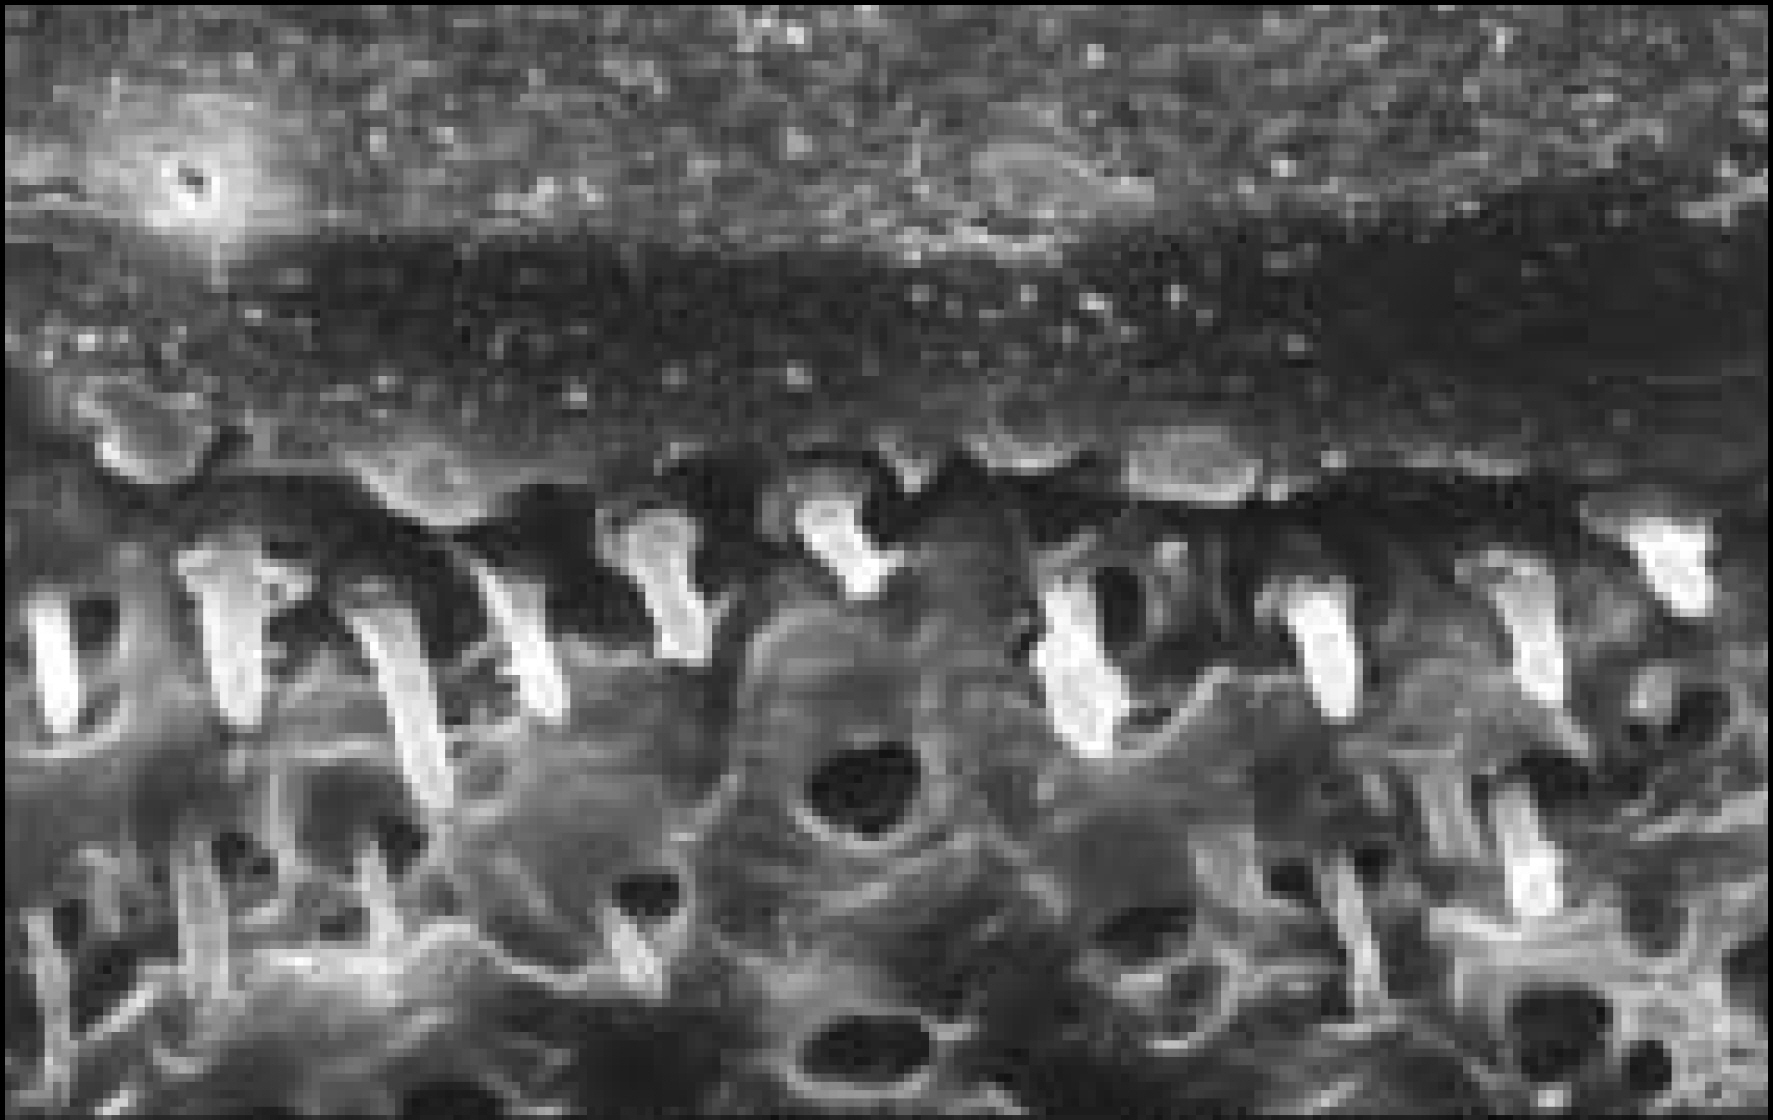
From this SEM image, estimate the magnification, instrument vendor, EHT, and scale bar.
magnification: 2 K X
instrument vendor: Hitachi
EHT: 20 kV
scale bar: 20000 nm